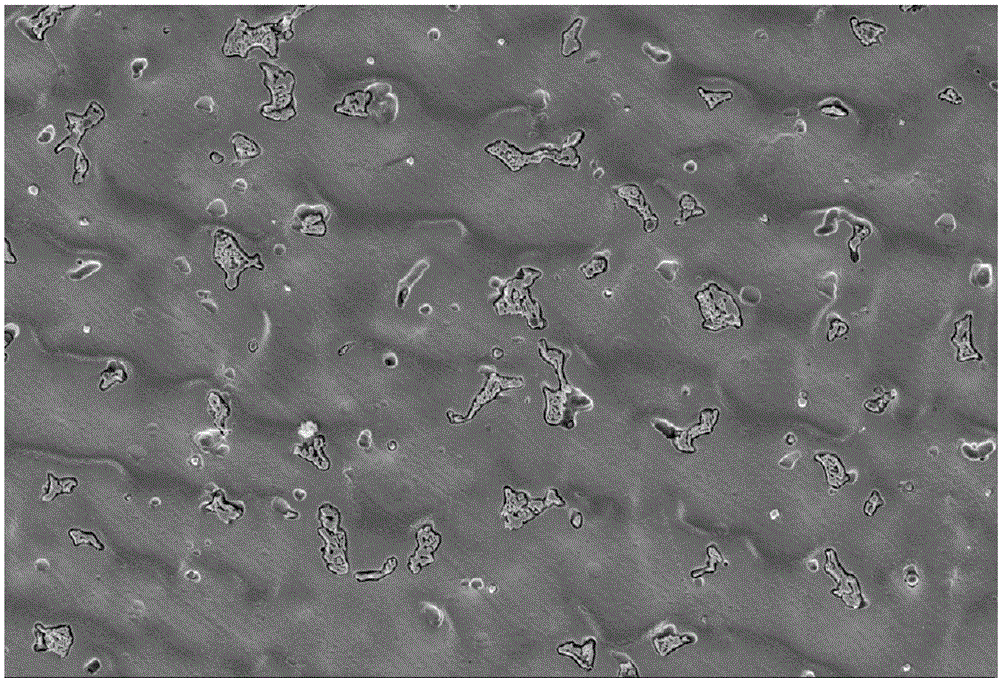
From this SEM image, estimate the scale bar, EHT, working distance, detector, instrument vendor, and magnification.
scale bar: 10000 nm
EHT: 15 kV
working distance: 14.1 mm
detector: SE2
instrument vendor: Zeiss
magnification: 1 K X